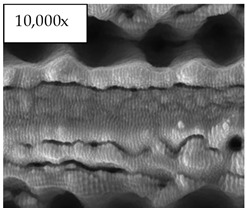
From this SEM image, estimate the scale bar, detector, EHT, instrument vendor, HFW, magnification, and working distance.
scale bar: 10000 nm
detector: ETD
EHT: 30 kV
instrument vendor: FEI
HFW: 29.8 µm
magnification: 10 K X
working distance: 7 mm